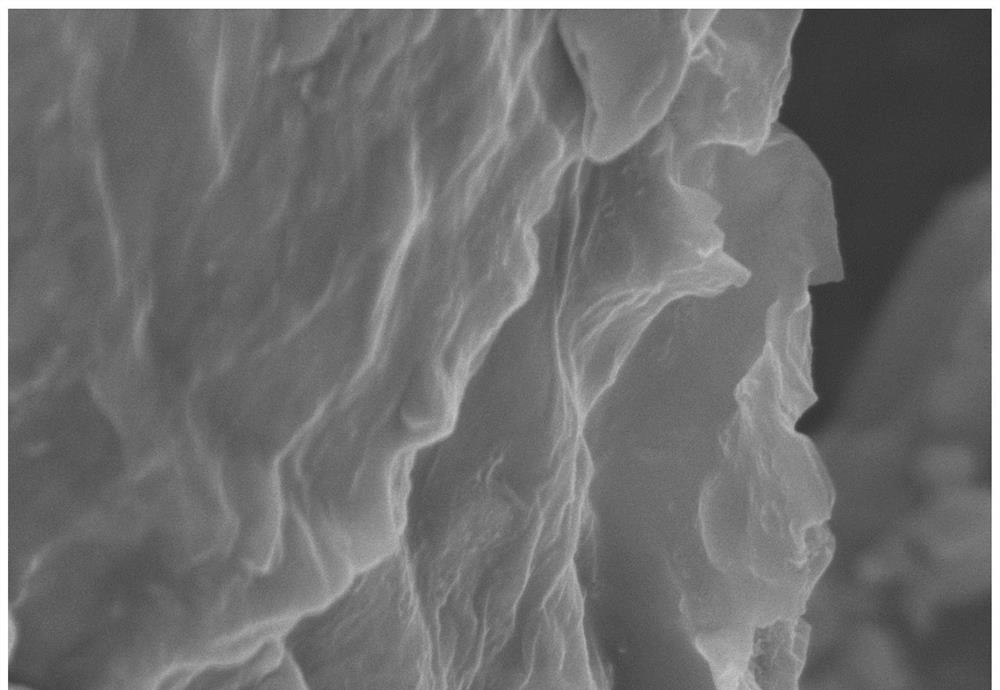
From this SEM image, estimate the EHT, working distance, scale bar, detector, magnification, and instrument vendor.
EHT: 5 kV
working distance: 9.5 mm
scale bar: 1000 nm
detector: SE(U)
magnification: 50 K X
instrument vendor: Hitachi